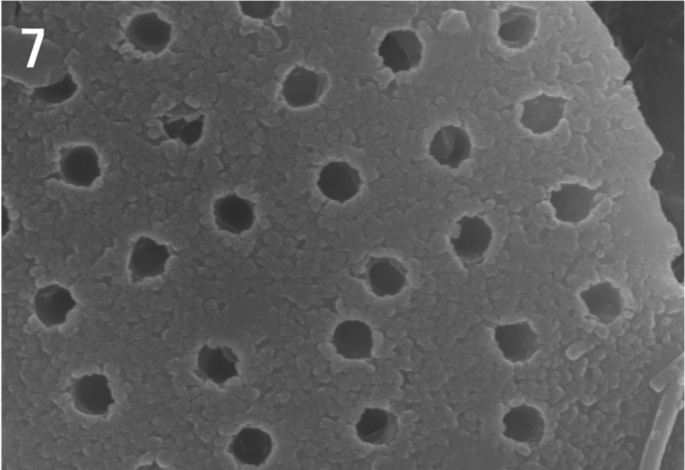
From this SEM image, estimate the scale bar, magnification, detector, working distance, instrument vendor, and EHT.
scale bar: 1000 nm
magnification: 30 K X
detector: SE(U)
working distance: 11.8 mm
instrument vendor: Hitachi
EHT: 15 kV